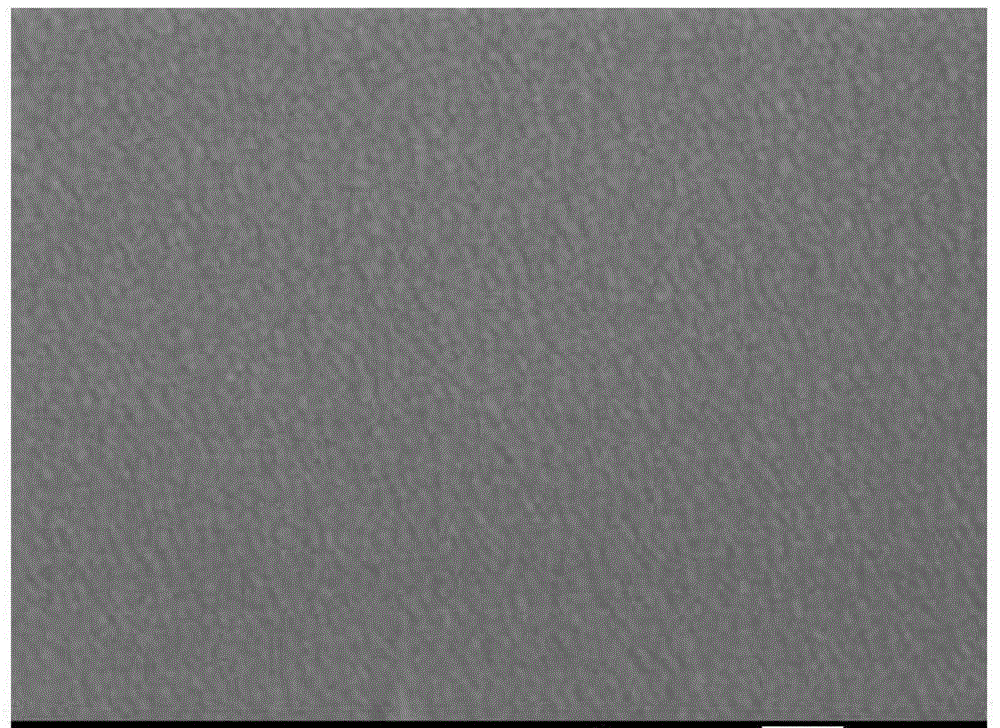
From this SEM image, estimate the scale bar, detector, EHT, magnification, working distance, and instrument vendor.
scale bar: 100 nm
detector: SEI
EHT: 15 kV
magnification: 100 K X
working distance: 15 mm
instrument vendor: JEOL